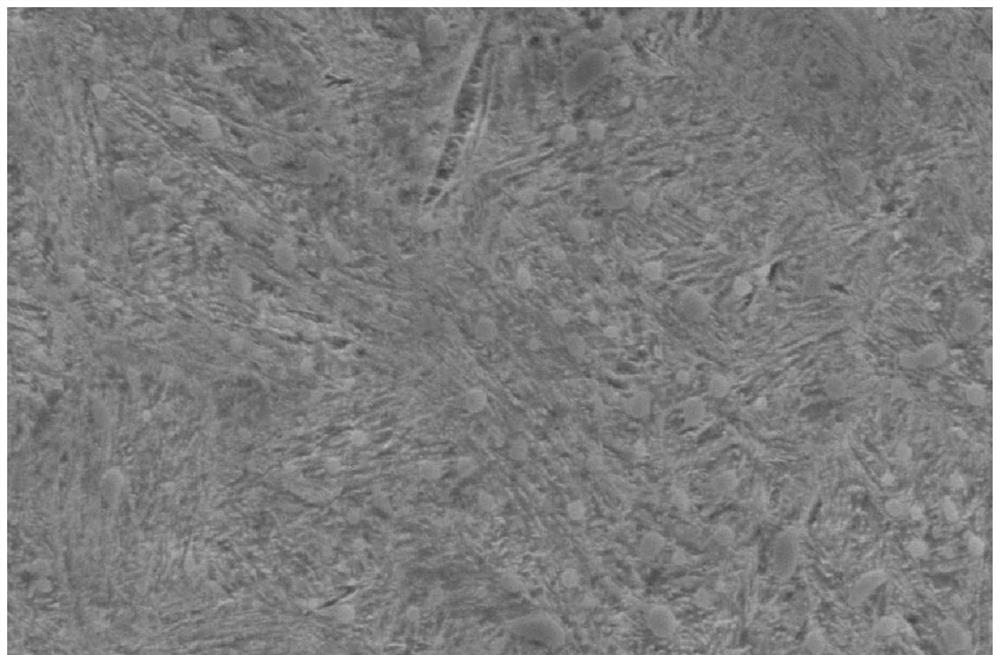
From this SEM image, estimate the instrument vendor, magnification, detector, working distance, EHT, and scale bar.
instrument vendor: JEOL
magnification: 4 K X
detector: SEI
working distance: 13 mm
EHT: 20 kV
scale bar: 5000 nm